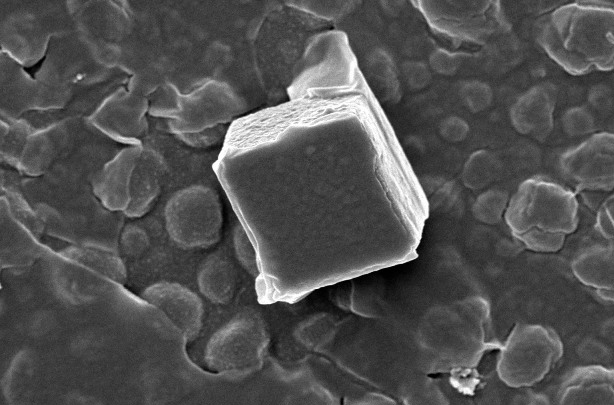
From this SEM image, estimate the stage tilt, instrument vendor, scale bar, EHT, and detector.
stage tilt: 0°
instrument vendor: FEI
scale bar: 4000 nm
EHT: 5 kV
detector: TLD